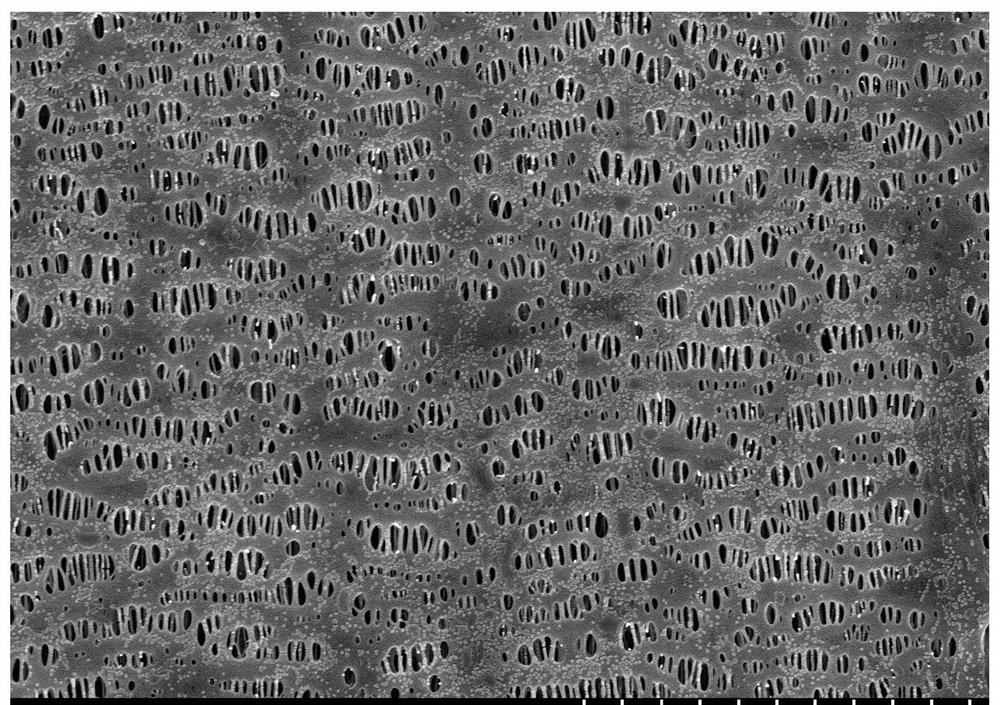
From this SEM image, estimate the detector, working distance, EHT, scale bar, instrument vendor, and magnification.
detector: SE(M)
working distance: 7.7 mm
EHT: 5 kV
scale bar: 5000 nm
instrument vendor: Hitachi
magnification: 10 K X